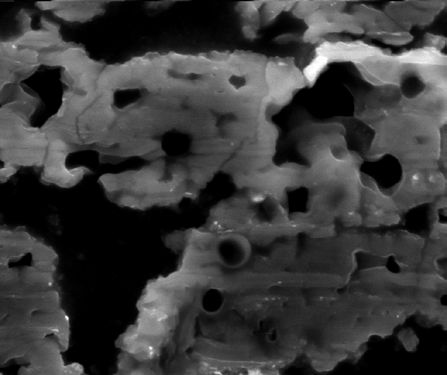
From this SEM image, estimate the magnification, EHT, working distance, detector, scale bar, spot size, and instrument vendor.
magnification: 2 K X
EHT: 30 kV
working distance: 12.4 mm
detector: ETD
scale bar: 20000 nm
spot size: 5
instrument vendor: FEI